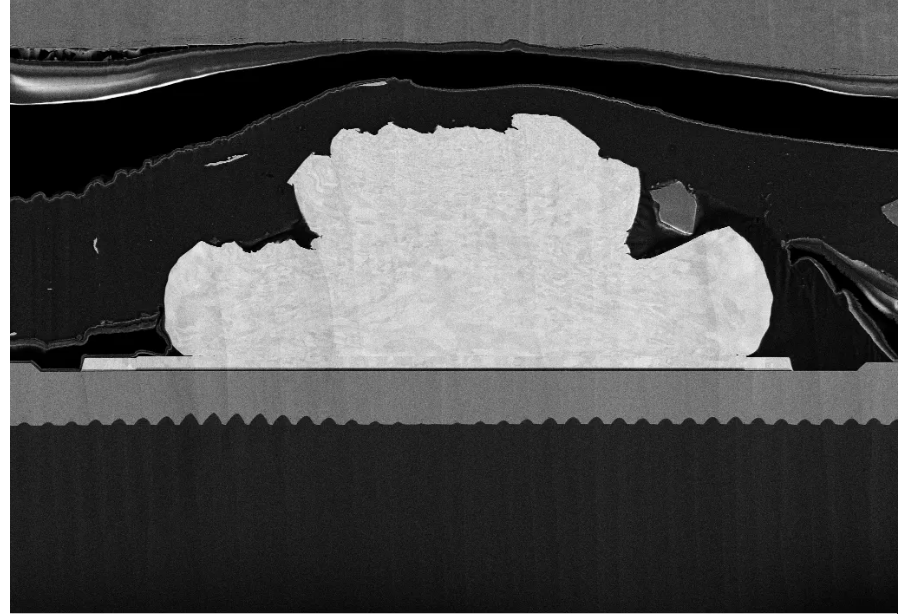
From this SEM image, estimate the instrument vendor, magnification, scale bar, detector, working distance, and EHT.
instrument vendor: Zeiss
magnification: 1 K X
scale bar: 10000 nm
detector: SE2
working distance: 4.9 mm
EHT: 5 kV